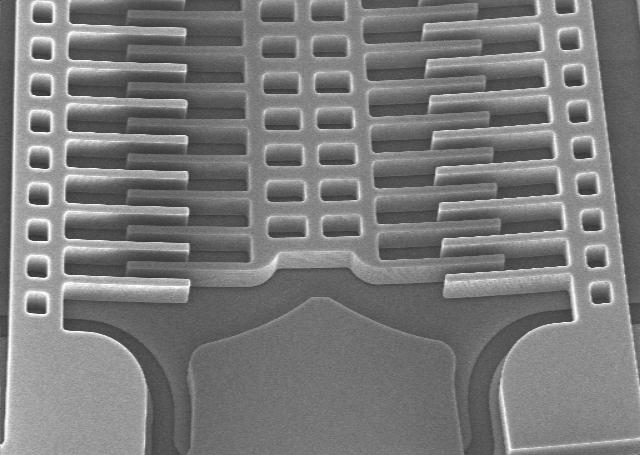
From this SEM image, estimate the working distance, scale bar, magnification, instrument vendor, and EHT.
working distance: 3 mm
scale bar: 20000 nm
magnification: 0.85 K X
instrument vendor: other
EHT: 10 kV